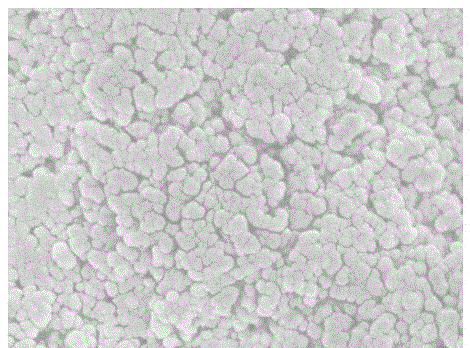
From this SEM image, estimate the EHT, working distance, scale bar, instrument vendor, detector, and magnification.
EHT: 3 kV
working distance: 5.3 mm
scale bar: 100 nm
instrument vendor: JEOL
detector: SEI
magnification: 30 K X